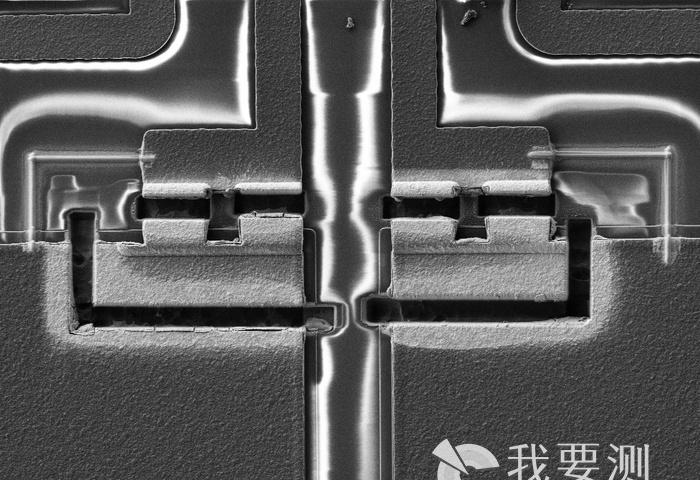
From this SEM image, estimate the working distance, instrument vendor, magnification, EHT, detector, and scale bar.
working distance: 4.1 mm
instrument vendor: Zeiss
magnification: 0.619 K X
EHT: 3 kV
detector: SE2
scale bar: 10000 nm